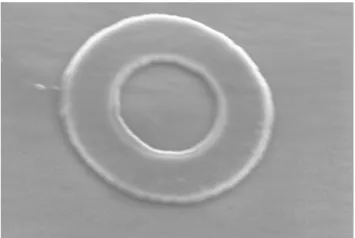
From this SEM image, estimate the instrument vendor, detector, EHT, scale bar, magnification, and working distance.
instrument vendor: Zeiss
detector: InLens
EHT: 5 kV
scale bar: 200 nm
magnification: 37.38 K X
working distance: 3.9 mm